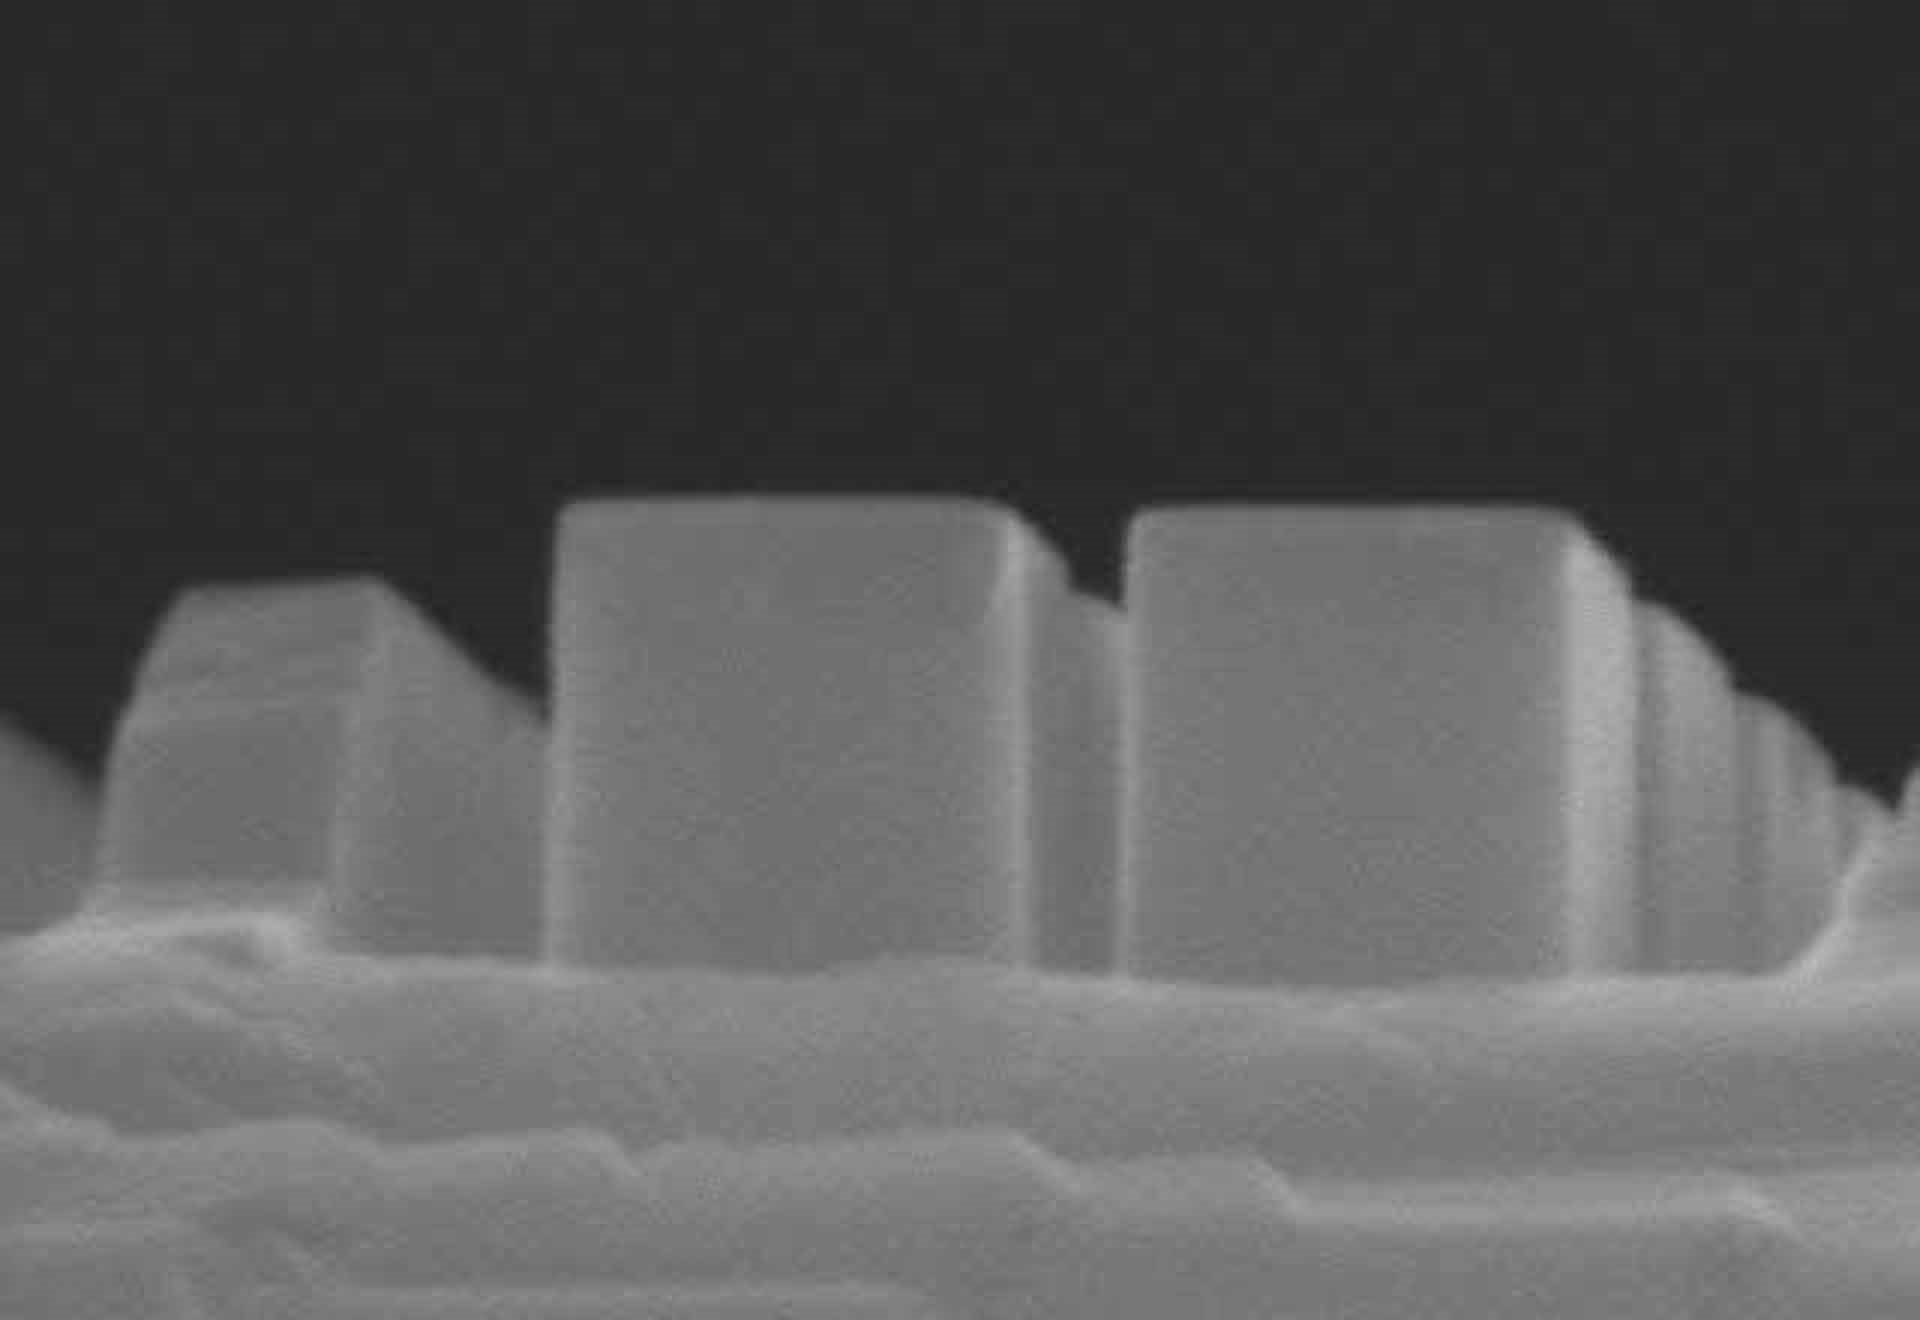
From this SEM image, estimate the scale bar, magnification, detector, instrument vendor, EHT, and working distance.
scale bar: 500 nm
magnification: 110 K X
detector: SE(M)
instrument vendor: Hitachi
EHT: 15 kV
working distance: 15.8 mm